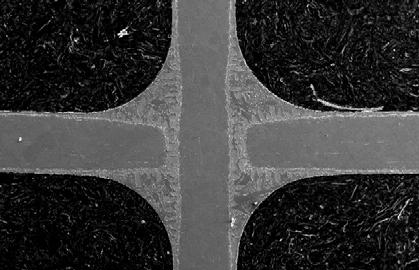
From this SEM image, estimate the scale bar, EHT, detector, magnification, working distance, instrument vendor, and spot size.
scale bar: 500000 nm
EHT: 20 kV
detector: SE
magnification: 0.1 K X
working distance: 10.7 mm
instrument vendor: FEI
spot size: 4